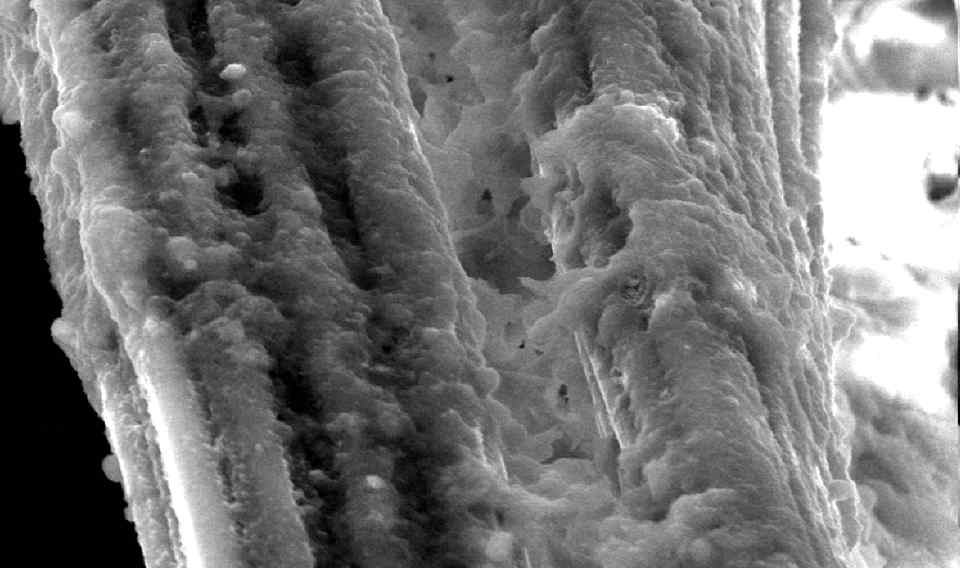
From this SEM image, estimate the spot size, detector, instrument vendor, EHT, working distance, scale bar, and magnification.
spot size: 3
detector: GSE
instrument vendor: FEI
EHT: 15 kV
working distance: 10.4 mm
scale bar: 10000 nm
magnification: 3 K X